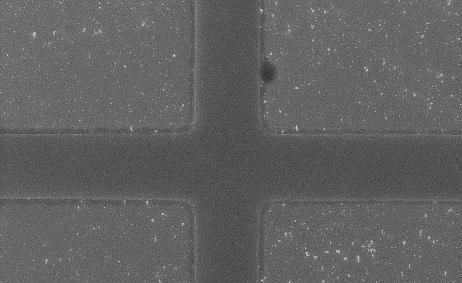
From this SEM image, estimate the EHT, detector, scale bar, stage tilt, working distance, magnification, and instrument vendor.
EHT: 15 kV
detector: InLens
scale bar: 10000 nm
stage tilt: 0°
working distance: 10.8 mm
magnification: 2 K X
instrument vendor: Zeiss